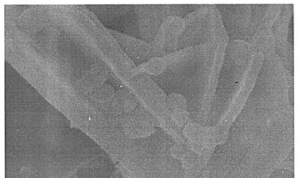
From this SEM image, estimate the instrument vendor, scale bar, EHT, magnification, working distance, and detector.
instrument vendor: Hitachi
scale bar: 500 nm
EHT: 10 kV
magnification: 100 K X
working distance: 10.8 mm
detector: SE(M)